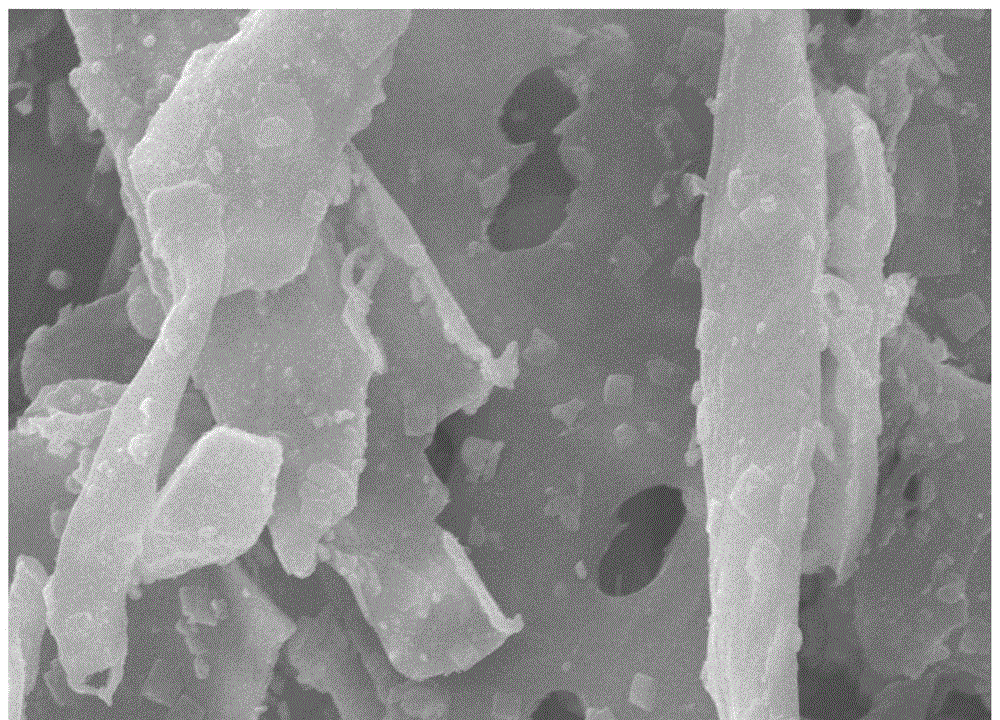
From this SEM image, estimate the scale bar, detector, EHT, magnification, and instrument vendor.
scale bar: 5000 nm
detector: SEI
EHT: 15 kV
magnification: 5 K X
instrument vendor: JEOL